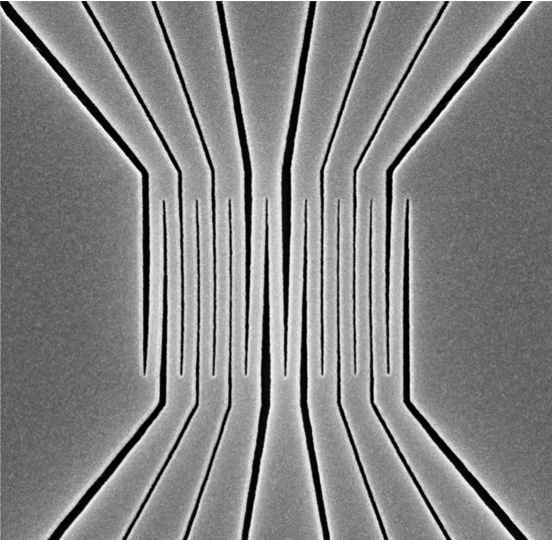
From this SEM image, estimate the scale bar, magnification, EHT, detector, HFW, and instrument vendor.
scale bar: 1000 nm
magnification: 50 K X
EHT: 10 kV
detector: SED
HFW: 5.37 µm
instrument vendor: other